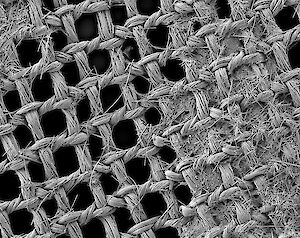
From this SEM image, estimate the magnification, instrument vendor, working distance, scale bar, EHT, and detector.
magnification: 0.033 K X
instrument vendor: JEOL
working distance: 12 mm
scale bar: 100000 nm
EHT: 3 kV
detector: LEI